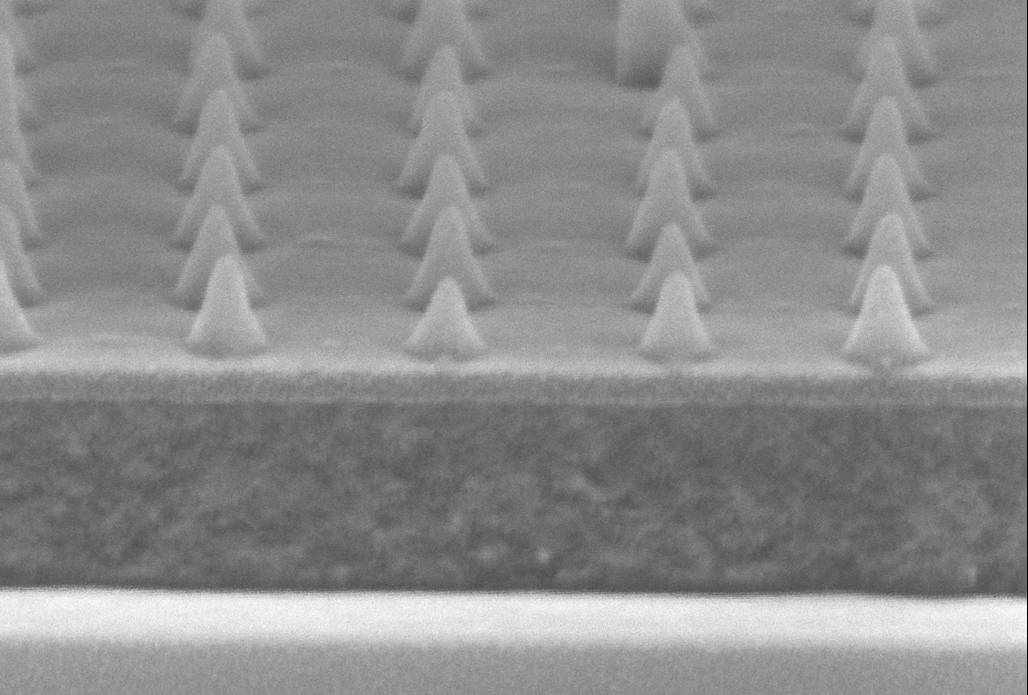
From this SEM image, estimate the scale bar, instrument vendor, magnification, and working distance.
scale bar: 100 nm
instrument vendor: Zeiss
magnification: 400 K X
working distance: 5 mm